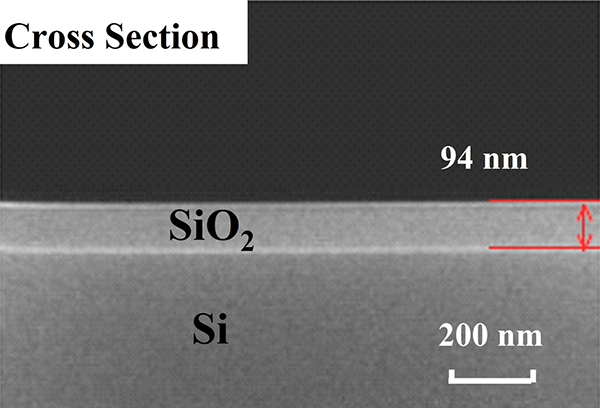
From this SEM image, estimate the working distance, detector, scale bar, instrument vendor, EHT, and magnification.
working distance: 12.9 mm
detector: SE(U)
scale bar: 500 nm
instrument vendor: Hitachi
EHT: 10 kV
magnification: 100 K X